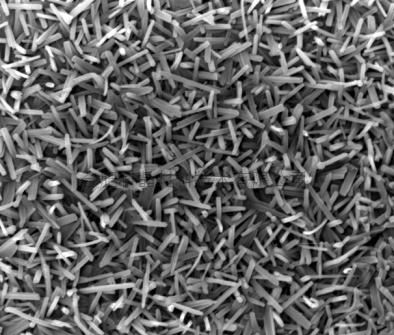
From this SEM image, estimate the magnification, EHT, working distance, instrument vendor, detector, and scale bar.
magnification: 20 K X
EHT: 20 kV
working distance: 6 mm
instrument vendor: FEI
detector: TLD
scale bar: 2000 nm